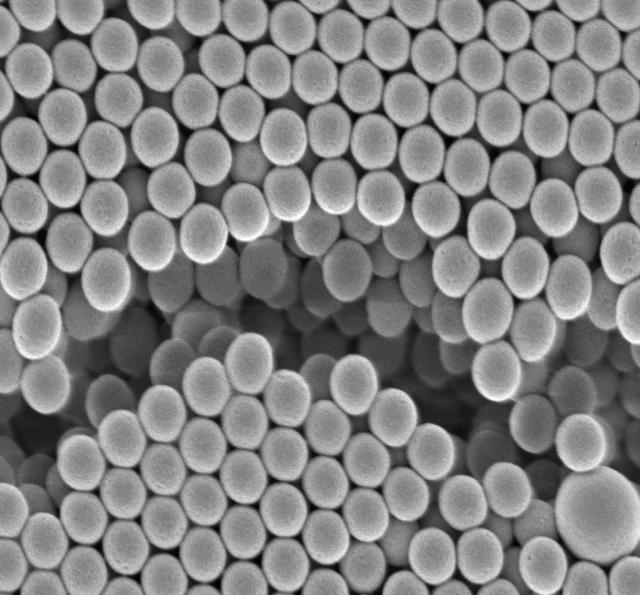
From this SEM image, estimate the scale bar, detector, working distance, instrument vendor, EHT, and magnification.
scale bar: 100 nm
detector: UED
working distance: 4 mm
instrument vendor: JEOL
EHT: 1 kV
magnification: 40 K X